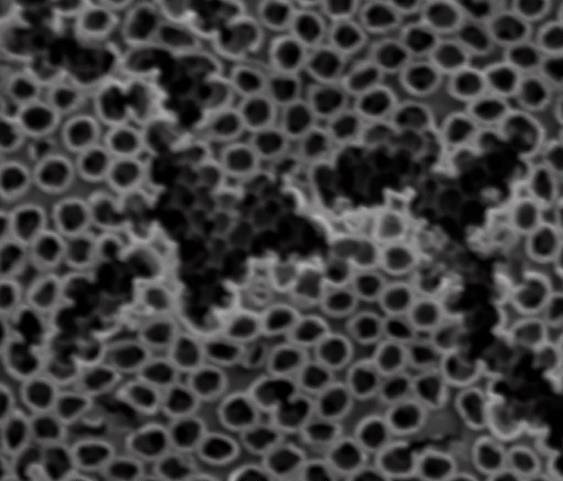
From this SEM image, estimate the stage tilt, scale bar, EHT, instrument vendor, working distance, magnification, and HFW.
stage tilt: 0°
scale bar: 2000 nm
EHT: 25 kV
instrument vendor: FEI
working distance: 10.9 mm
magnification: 50 K X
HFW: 5.41 µm